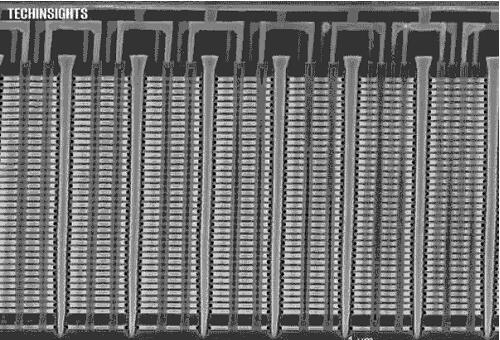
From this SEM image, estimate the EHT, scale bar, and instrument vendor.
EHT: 5 kV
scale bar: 1000 nm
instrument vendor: Zeiss